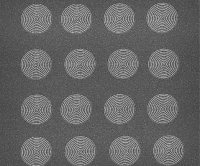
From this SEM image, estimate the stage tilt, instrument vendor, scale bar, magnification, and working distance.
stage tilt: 0.1°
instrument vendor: FEI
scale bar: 10000 nm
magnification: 10 K X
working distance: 7.2 mm